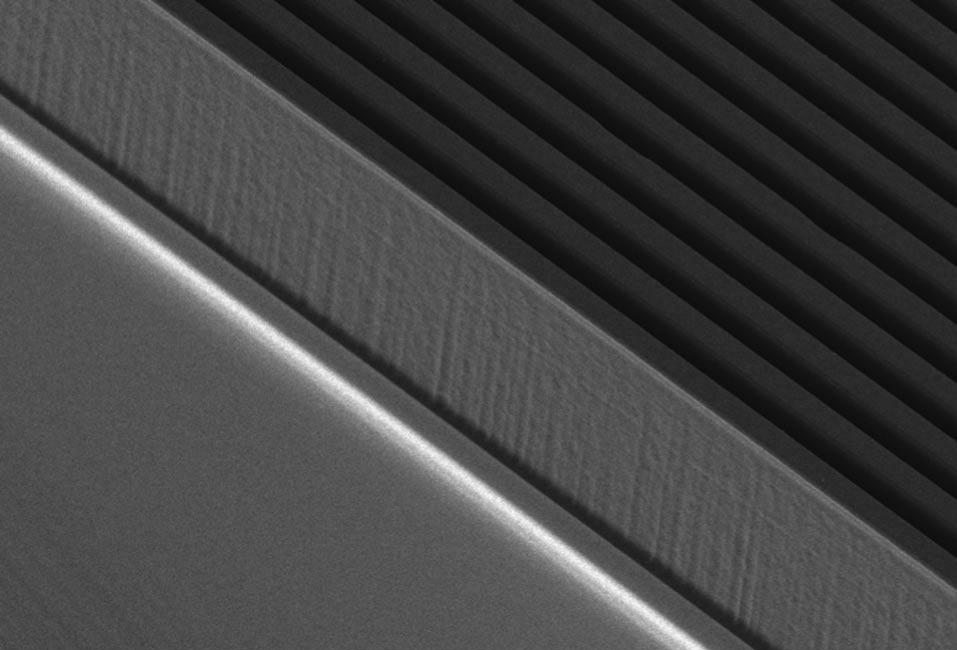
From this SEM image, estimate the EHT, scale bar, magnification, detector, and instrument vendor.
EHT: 3 kV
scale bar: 20000 nm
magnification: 0.9 K X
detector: SEI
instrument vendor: JEOL